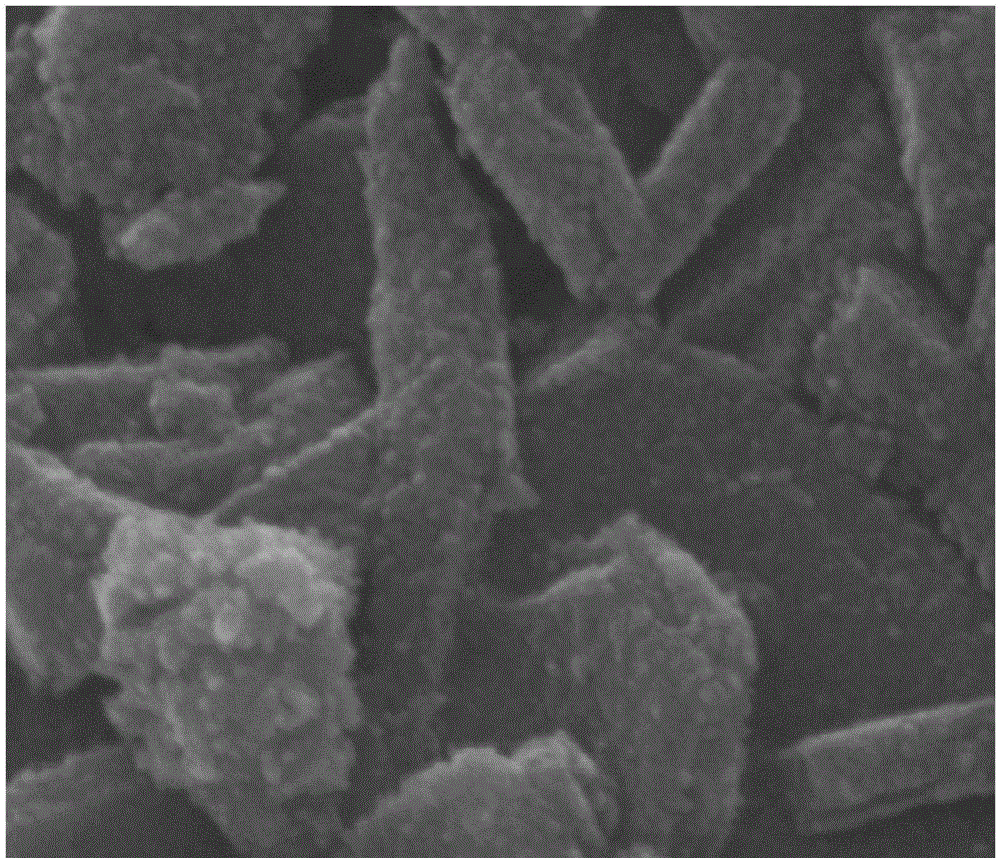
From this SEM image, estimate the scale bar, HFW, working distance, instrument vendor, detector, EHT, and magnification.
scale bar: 300 nm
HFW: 1.35 µm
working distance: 9.7 mm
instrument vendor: FEI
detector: ETD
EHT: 20 kV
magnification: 100 K X